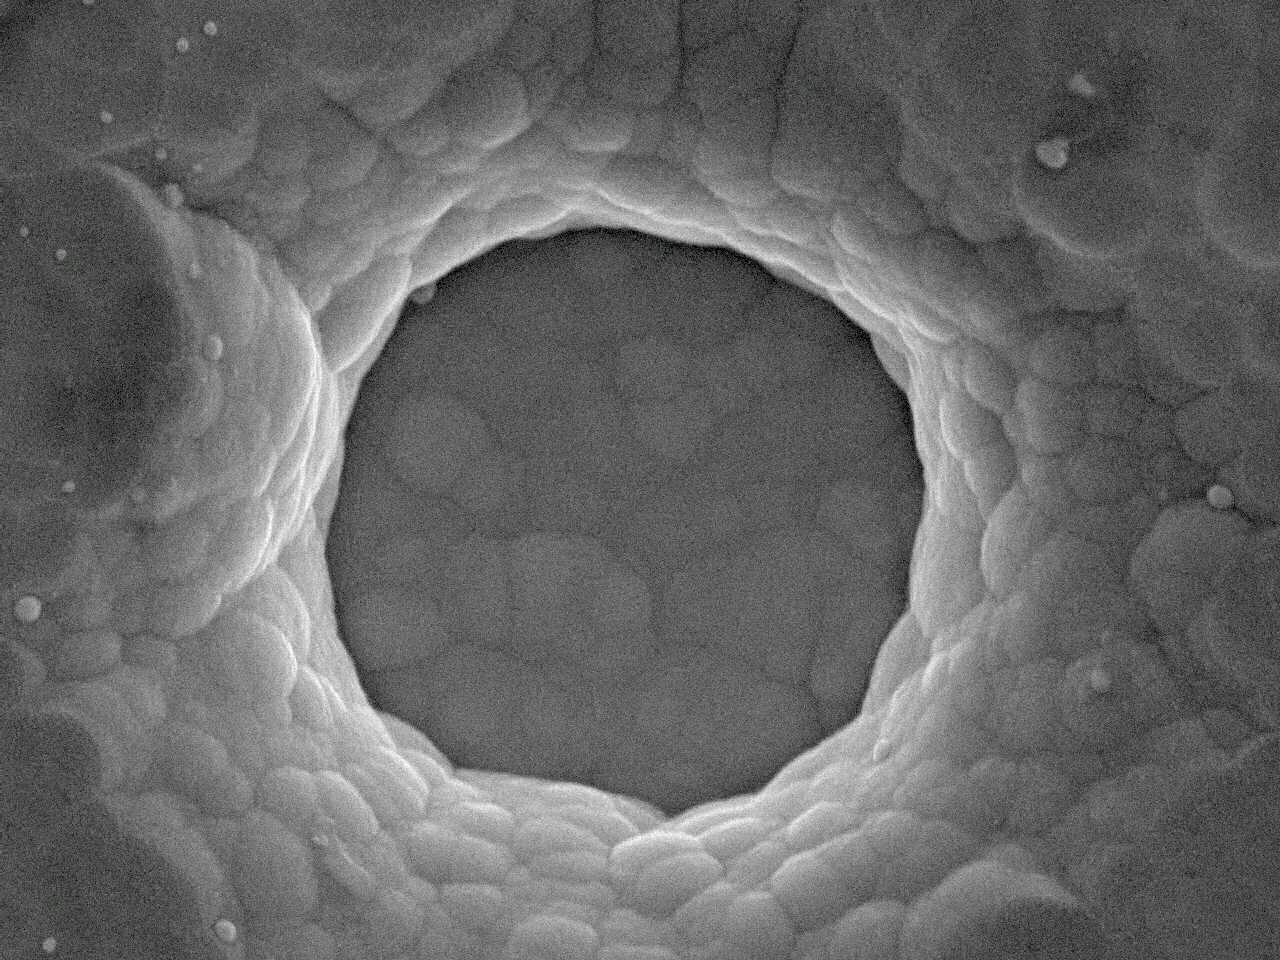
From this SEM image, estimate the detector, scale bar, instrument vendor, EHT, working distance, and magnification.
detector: SEI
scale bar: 1000 nm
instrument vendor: JEOL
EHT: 5 kV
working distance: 10 mm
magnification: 20 K X